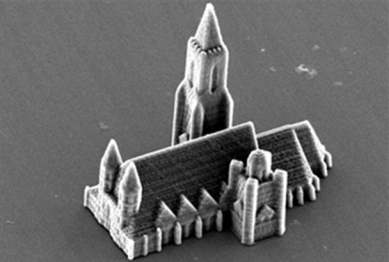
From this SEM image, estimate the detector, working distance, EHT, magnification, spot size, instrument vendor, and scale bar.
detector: SE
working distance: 26.8 mm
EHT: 12 kV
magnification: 0.817 K X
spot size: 4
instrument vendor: FEI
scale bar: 20000 nm